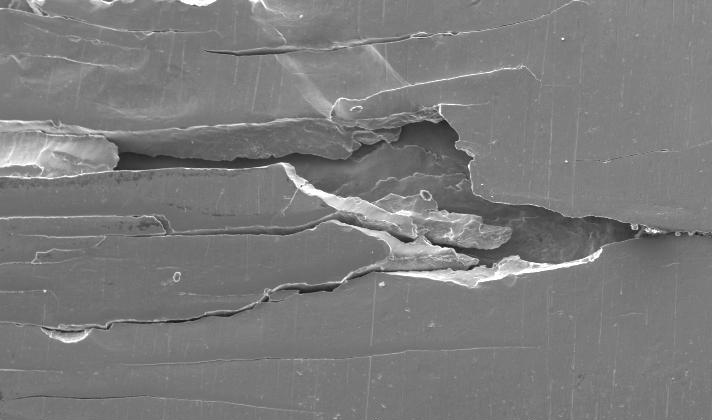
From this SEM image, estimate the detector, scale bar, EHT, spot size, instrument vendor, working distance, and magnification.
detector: SE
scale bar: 200000 nm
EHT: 20 kV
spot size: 4.8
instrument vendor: FEI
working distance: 11.9 mm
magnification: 0.1 K X